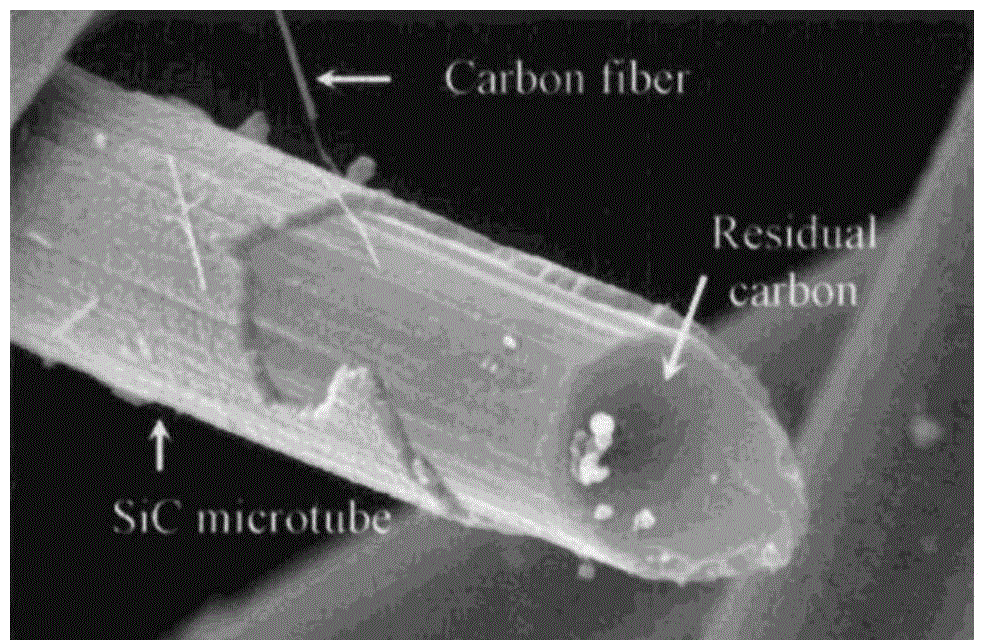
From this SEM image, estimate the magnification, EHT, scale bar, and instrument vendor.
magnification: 5 K X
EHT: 20 kV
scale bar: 5000 nm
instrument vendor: JEOL